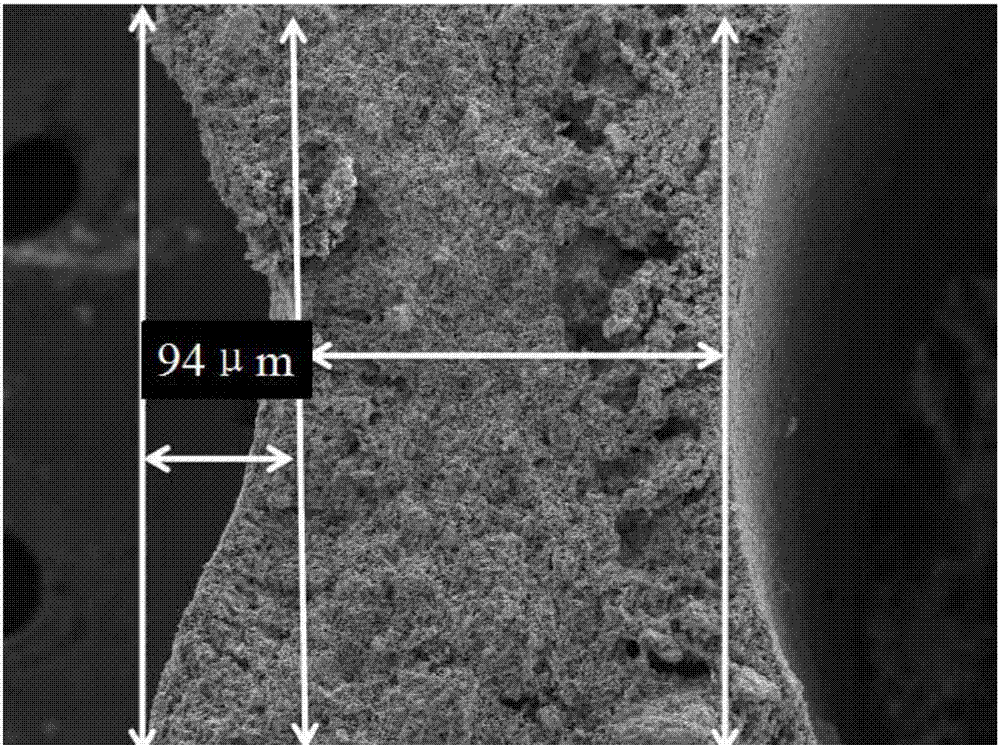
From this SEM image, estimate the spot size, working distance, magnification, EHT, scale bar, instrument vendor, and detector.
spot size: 3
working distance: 5 mm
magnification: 0.2 K X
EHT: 10 kV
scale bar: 100000 nm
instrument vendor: FEI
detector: SE